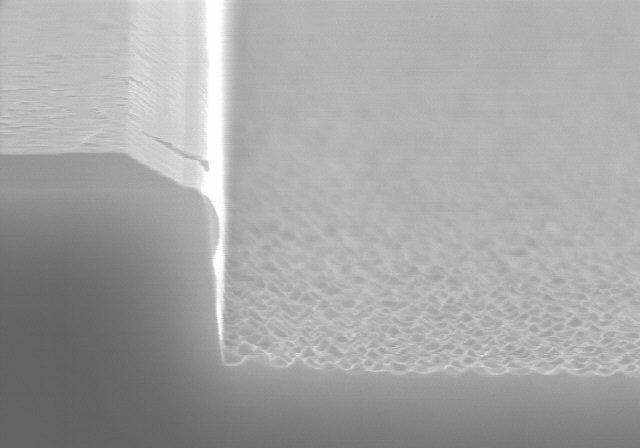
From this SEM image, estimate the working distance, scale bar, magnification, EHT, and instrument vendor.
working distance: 11.7 mm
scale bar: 1000 nm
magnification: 30 K X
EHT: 15 kV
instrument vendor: Hitachi